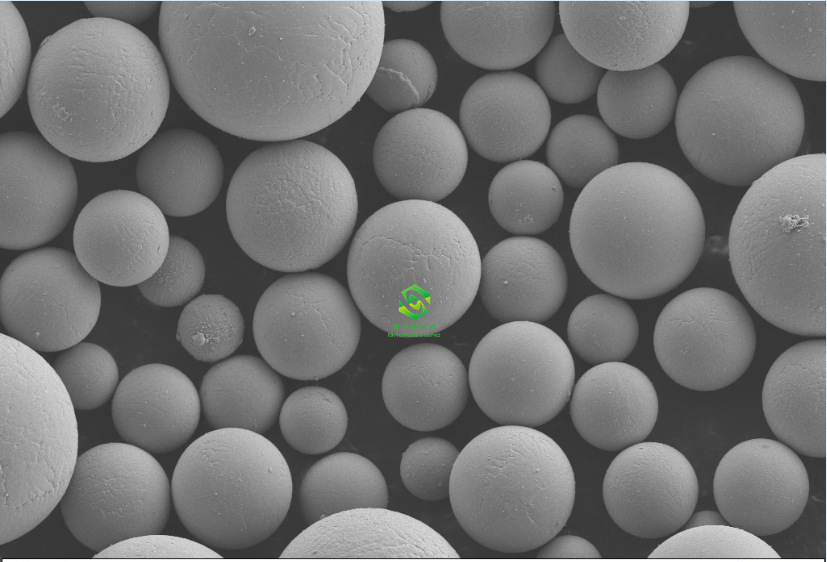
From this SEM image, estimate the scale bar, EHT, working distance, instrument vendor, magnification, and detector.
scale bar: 10000 nm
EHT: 5 kV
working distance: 7.5 mm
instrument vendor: Zeiss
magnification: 1 K X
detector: SE2